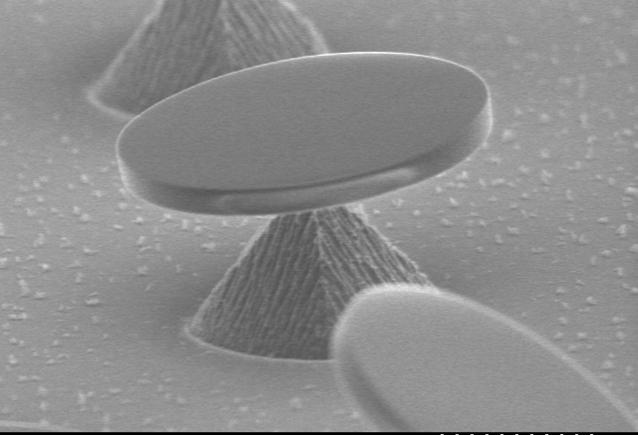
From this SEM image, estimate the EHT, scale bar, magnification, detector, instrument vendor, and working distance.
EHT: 5 kV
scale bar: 20000 nm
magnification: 1.5 K X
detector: SE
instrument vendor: JEOL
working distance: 20.4 mm